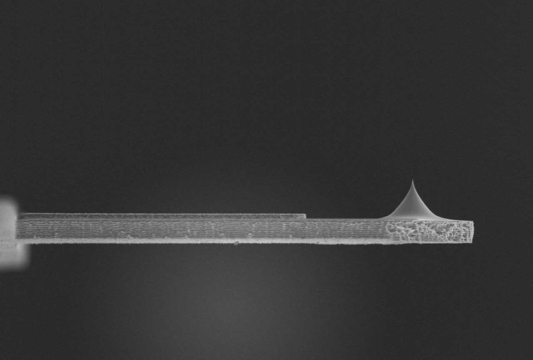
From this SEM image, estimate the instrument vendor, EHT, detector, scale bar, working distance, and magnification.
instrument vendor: Hitachi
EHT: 4 kV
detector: SE(UL)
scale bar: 40000 nm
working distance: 8.9 mm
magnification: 1.3 K X